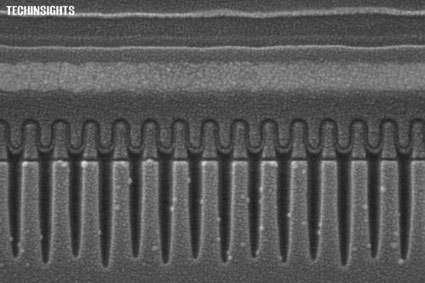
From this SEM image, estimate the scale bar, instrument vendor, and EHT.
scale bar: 100 nm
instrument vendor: Zeiss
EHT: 5 kV